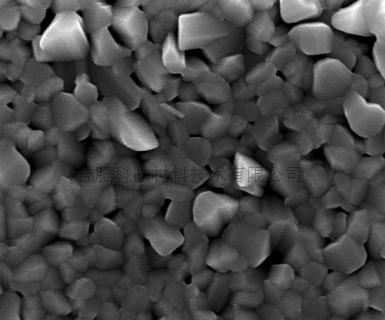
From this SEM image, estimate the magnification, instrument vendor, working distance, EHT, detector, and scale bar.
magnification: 20 K X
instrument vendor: FEI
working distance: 6 mm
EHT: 20 kV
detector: TLD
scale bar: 2000 nm